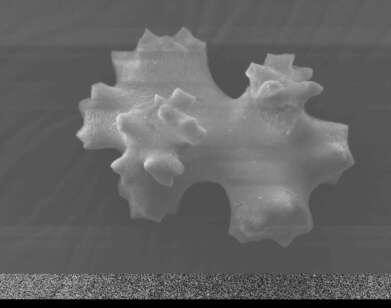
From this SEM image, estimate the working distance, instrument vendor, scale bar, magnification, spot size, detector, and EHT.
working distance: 20.1 mm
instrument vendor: FEI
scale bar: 20000 nm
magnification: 3 K X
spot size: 3.5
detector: SE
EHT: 12.5 kV